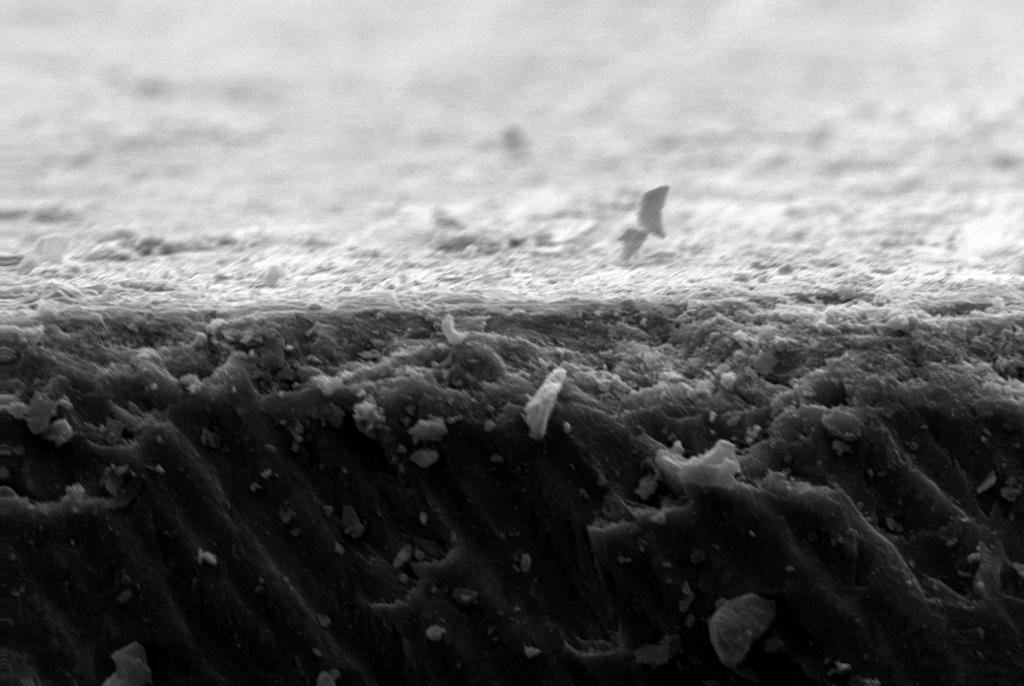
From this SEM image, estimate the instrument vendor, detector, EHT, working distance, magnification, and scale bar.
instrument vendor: Zeiss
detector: SE1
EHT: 20 kV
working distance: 7 mm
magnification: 1 K X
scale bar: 10000 nm